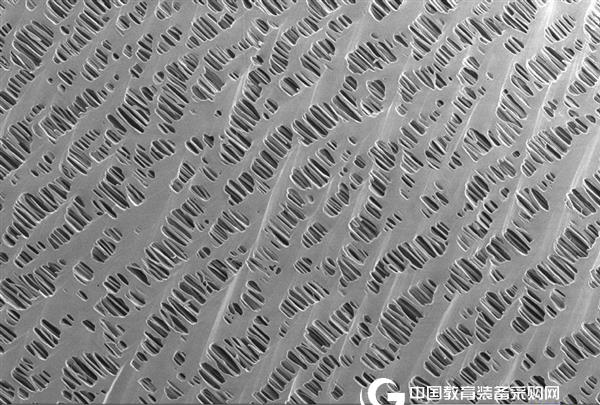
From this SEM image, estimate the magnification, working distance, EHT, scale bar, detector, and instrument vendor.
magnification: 10 K X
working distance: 1.7 mm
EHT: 0.5 kV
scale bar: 1000 nm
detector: InLens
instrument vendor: Zeiss